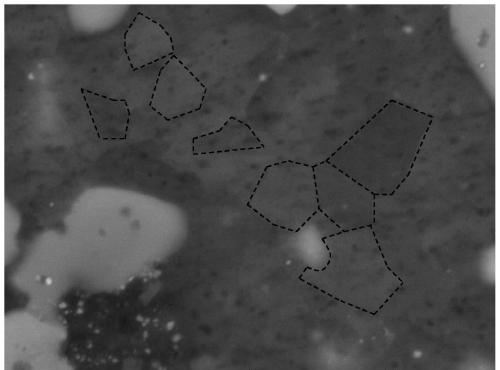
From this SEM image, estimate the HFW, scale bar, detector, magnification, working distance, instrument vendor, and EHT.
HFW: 3.46 µm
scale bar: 1000 nm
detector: BSE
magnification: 80 K X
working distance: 9.01 mm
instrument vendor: Tescan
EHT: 8 kV